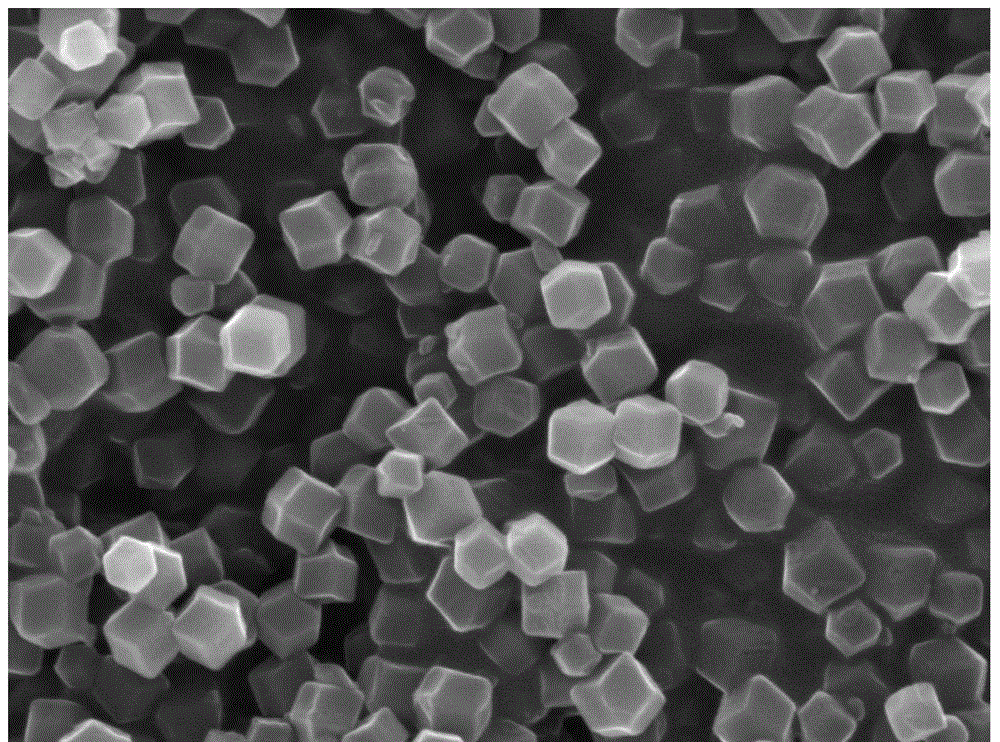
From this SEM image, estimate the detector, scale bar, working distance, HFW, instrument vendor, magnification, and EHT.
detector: SE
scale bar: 1000 nm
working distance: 18.07 mm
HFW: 4.61 µm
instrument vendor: Tescan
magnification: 60 K X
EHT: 15 kV